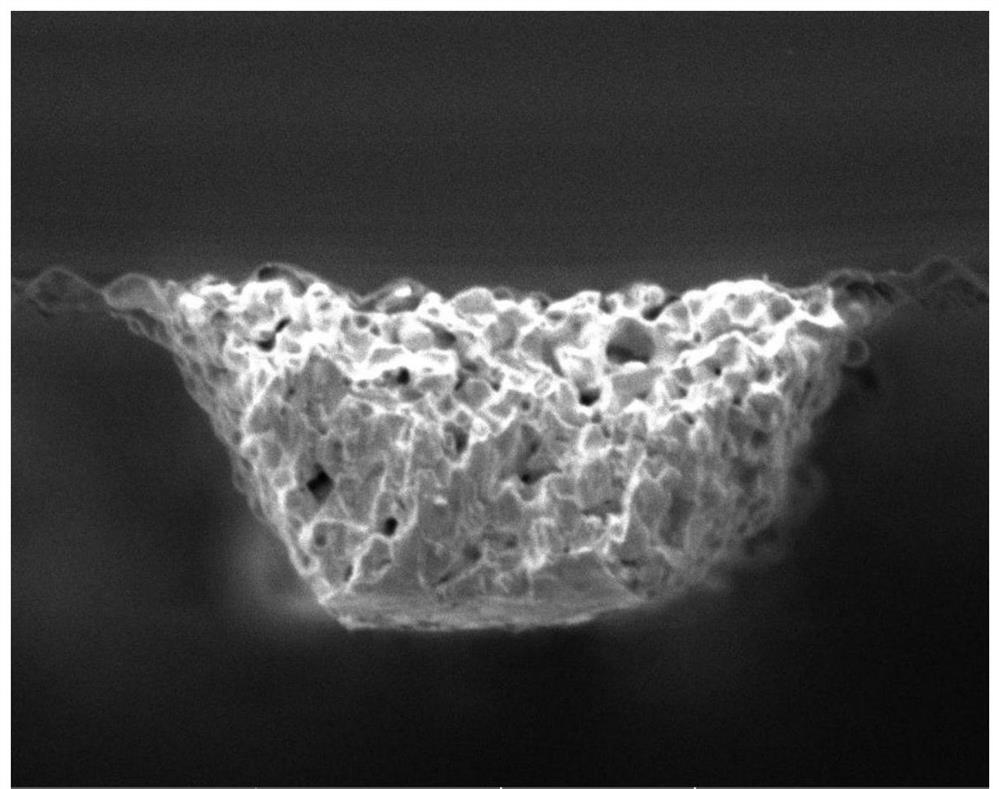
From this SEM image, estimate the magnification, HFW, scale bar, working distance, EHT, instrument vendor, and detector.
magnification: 5.49 K X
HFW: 50.4 µm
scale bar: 10000 nm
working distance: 17.34 mm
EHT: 5 kV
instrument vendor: Tescan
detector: SE